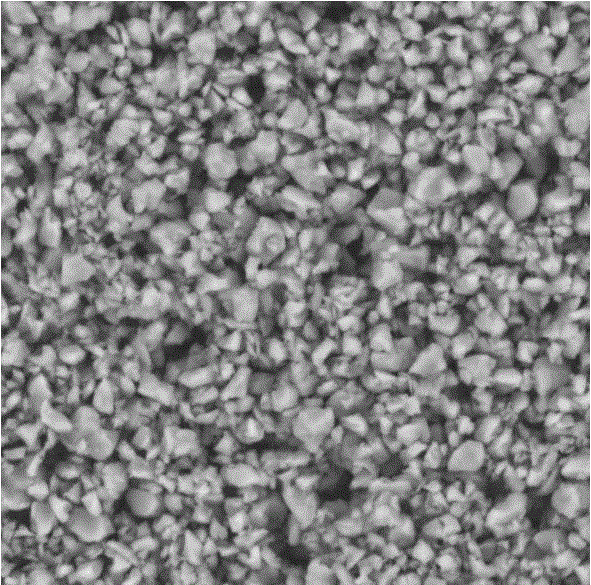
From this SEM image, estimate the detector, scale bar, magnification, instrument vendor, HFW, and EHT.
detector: BSD Full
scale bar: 5000 nm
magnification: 14 K X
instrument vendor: other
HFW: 19.2 µm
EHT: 10 kV